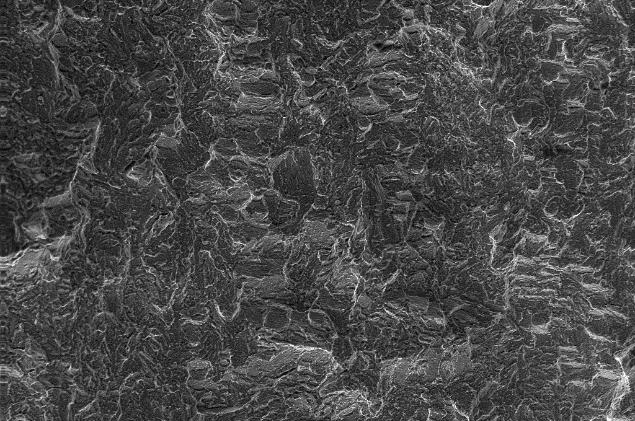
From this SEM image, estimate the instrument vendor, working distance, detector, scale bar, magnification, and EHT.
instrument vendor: Zeiss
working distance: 23 mm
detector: SE1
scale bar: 200000 nm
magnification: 0.2 K X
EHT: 20 kV